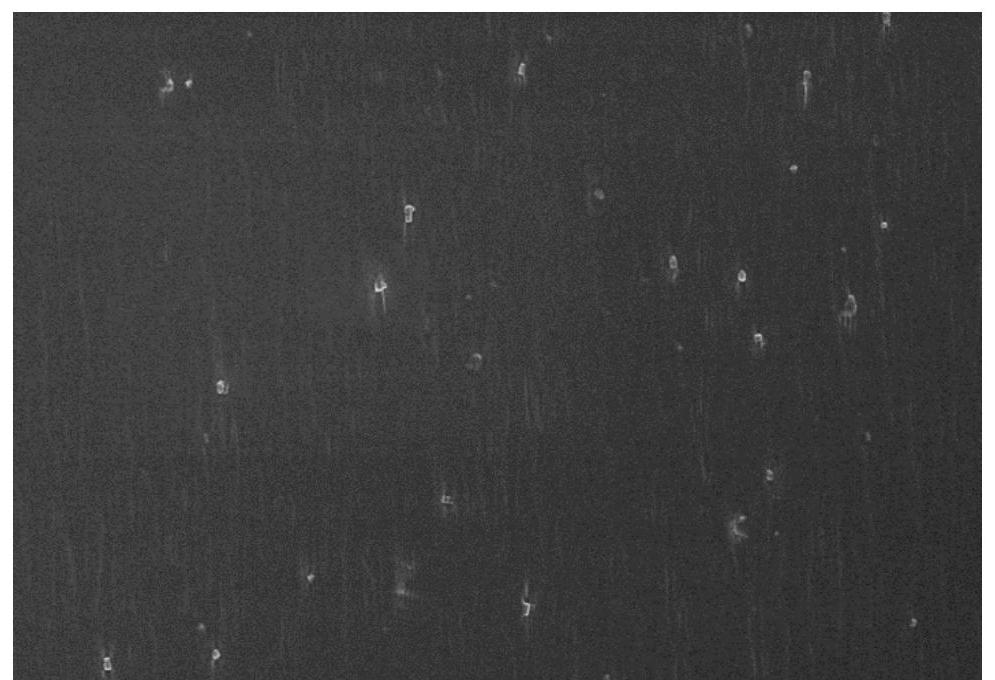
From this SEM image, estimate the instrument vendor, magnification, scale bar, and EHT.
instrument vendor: Hitachi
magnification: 1 K X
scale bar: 50000 nm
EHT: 5 kV